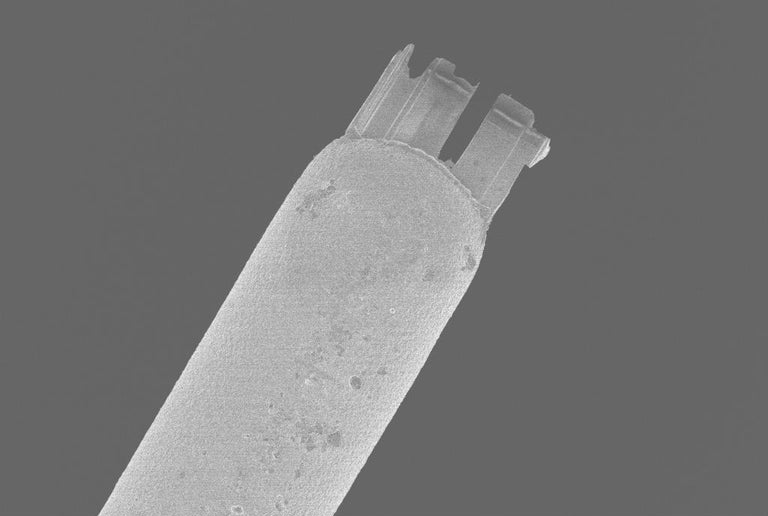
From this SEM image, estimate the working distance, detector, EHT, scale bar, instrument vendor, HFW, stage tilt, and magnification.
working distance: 4.9 mm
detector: InLens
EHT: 2 kV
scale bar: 10000 nm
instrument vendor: Zeiss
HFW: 243.8 µm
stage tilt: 0°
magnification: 0.469 K X